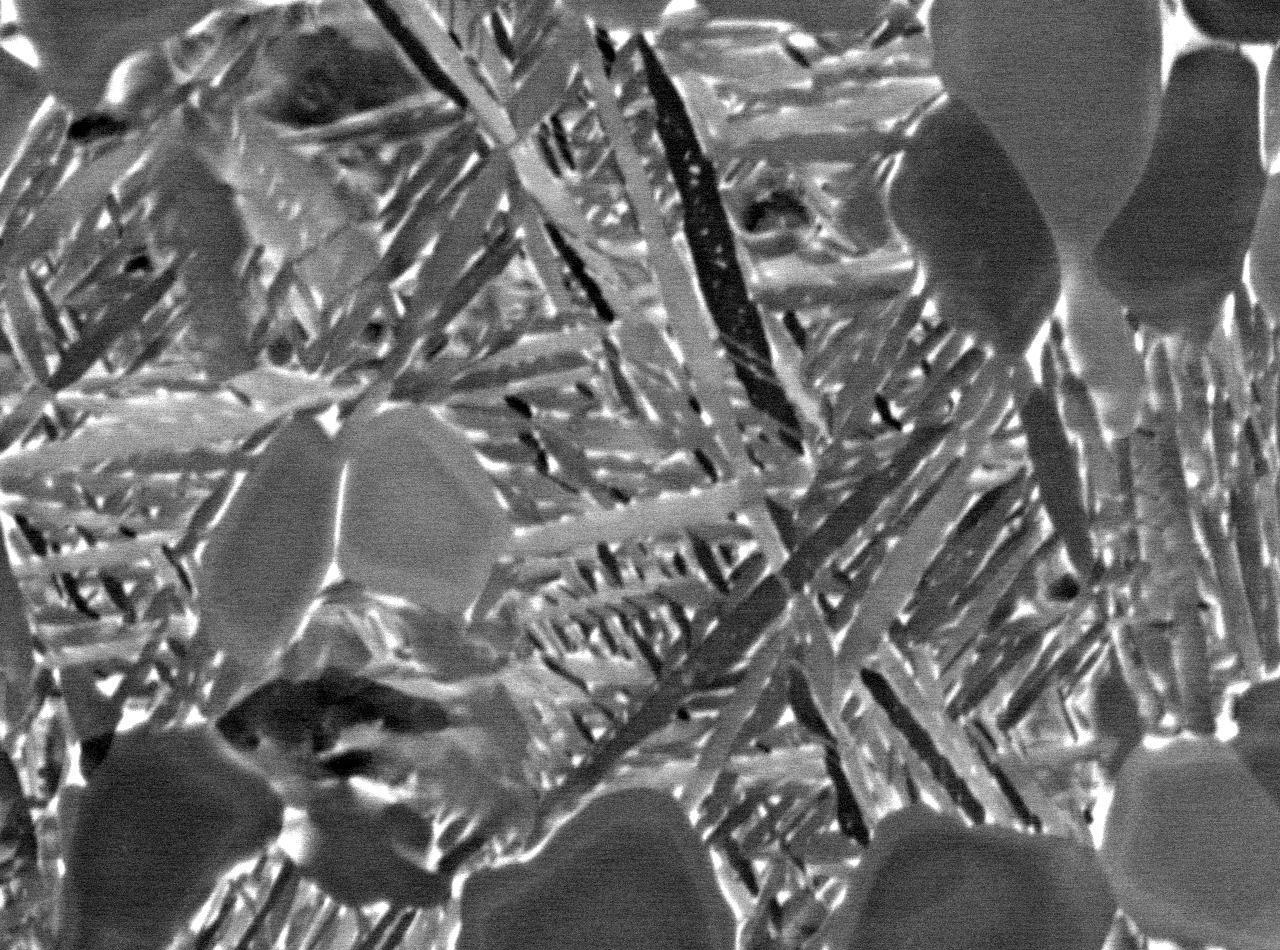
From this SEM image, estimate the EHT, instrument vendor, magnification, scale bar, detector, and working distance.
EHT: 15 kV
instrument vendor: JEOL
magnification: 5 K X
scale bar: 1000 nm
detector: COMPO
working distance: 10 mm